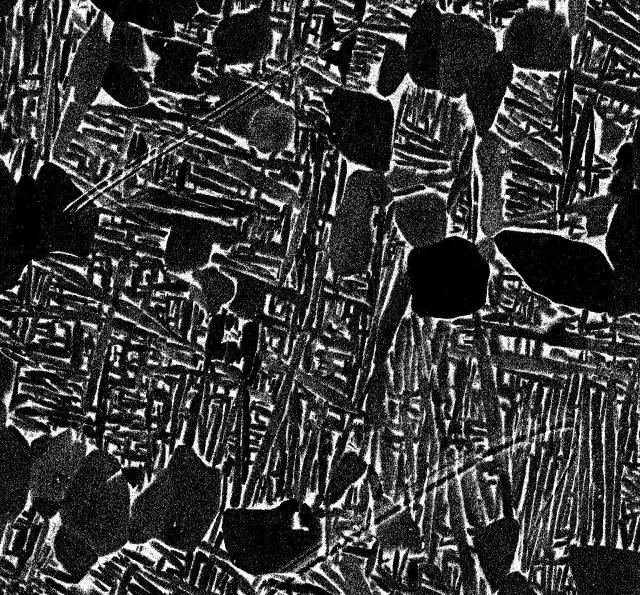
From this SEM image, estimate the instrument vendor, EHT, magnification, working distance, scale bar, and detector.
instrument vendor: JEOL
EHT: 15 kV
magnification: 2 K X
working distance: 10.1 mm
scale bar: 10000 nm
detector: COMPO